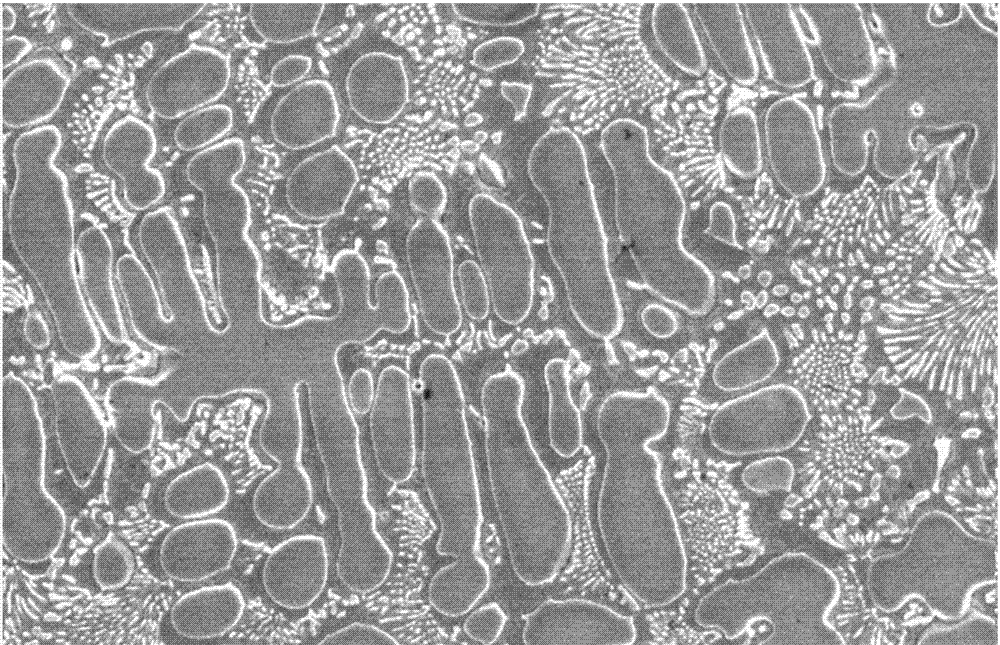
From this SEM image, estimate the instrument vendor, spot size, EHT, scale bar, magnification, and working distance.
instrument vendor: other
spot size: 3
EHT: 2 kV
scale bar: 5000 nm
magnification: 8 K X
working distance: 7.9 mm